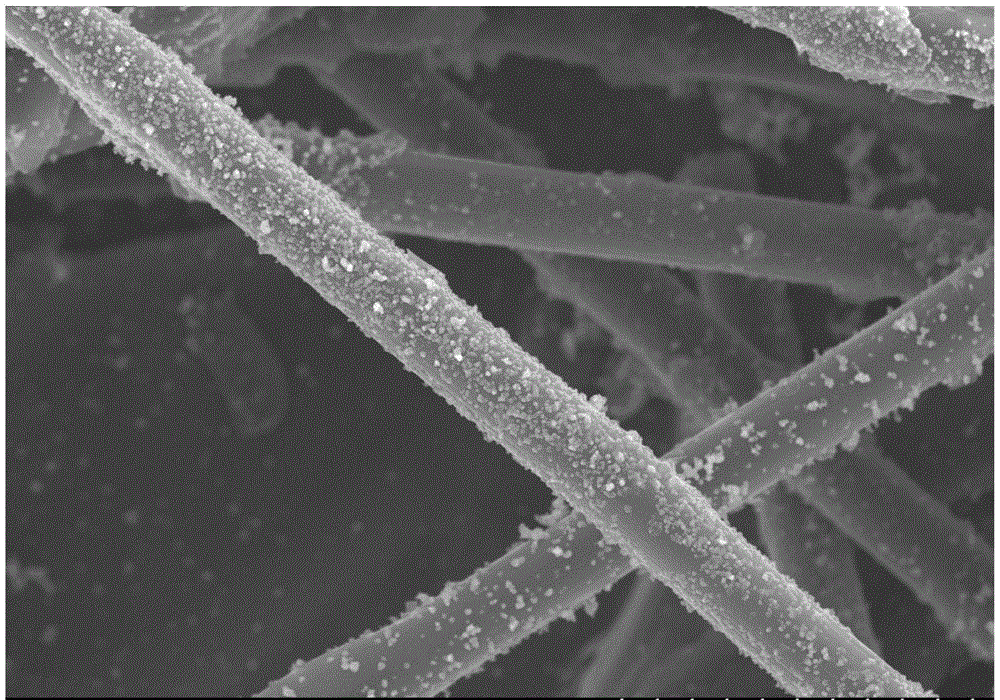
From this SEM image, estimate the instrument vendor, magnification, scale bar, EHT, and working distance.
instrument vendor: Hitachi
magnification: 1.5 K X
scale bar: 30000 nm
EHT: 20 kV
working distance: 11.8 mm